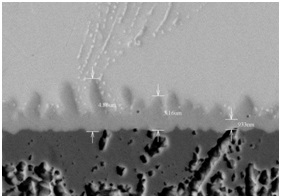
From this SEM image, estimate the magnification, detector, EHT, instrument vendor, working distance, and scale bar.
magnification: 5 K X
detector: BSE3D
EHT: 15 kV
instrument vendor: Hitachi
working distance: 8.9 mm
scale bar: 10000 nm